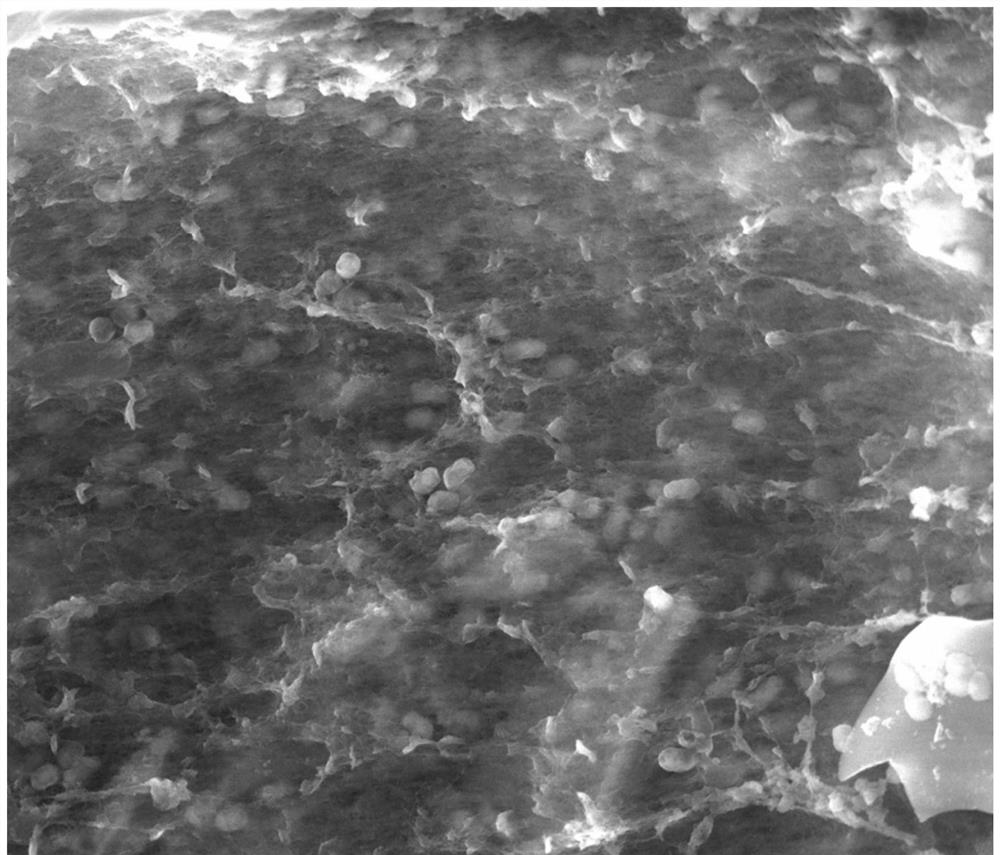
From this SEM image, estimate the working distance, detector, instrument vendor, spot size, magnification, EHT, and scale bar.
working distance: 10.2 mm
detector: LFD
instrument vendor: FEI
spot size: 3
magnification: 10 K X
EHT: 20 kV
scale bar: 10000 nm